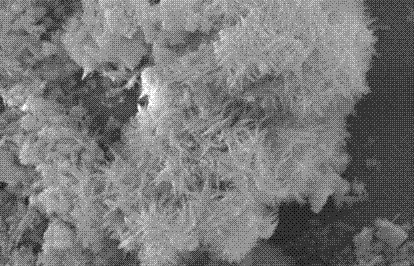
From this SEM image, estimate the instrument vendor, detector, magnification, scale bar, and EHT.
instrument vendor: JEOL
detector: SEI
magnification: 10 K X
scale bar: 1000 nm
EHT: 10 kV